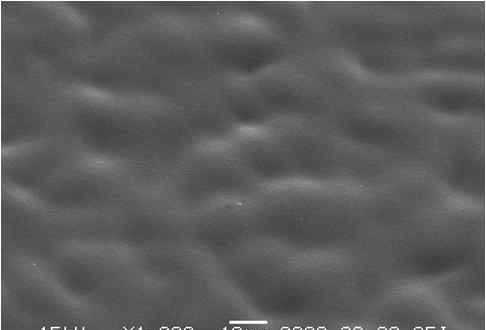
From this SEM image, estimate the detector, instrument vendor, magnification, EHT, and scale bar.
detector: SEI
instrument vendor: JEOL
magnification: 1 K X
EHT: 15 kV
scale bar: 10000 nm